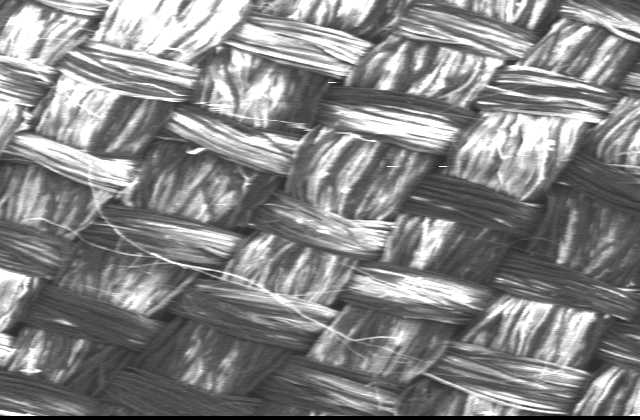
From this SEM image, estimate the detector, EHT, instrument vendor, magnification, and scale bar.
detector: SEI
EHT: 13 kV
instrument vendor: JEOL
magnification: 0.035 K X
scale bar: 500000 nm